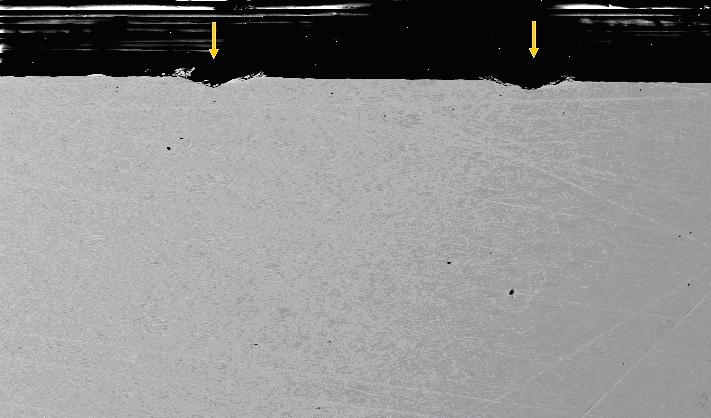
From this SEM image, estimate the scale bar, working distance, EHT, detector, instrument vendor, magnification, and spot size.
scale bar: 200000 nm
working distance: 9.9 mm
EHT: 20 kV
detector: BSE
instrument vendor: FEI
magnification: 0.2 K X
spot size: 6